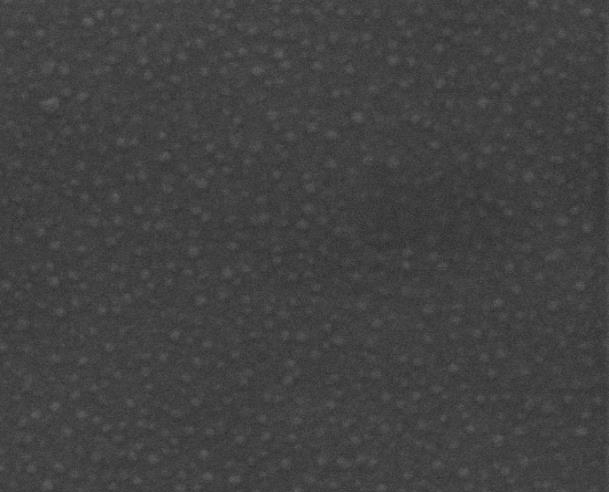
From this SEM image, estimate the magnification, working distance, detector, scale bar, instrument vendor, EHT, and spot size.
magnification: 160 K X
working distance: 10.7 mm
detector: ETD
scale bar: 500 nm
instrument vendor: FEI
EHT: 20 kV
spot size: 3